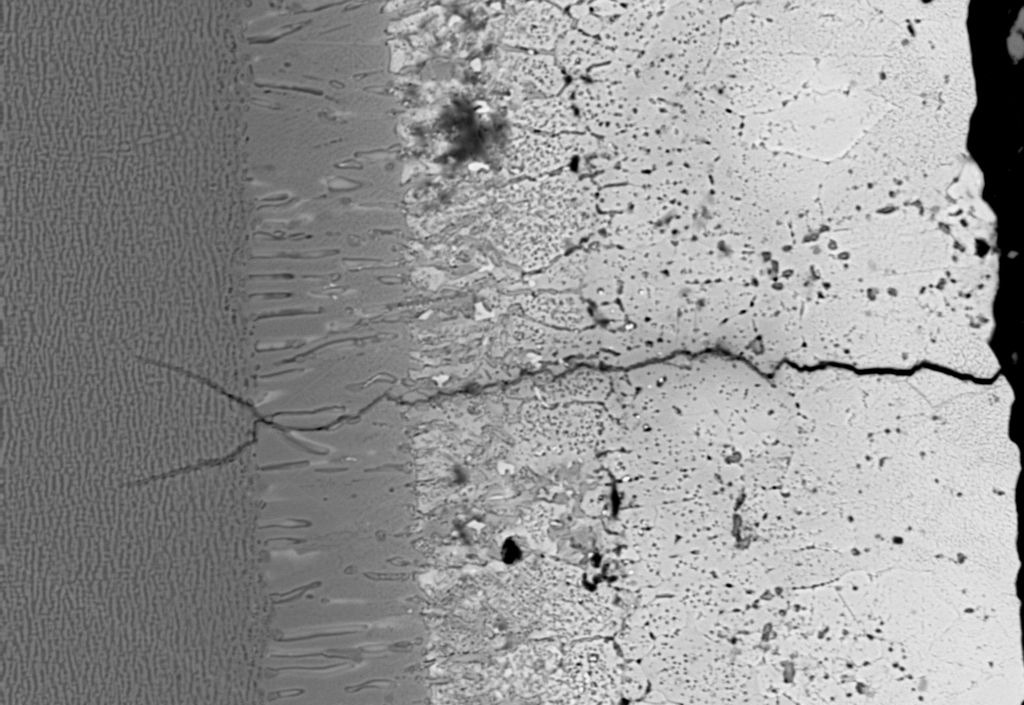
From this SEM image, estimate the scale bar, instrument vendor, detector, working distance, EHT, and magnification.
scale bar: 10000 nm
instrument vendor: Zeiss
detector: QBSD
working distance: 19 mm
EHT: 15 kV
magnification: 1 K X